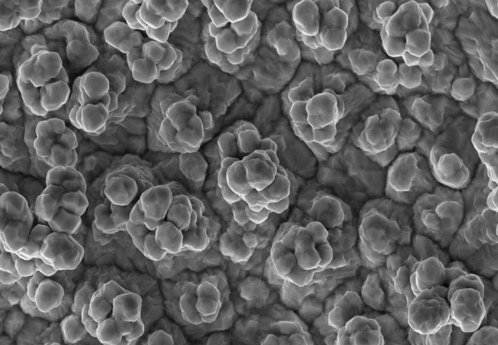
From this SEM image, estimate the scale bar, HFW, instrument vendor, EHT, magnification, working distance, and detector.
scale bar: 5000 nm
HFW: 25.4 µm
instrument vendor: Thermo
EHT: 15 kV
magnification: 5 K X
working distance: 10 mm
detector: ETD